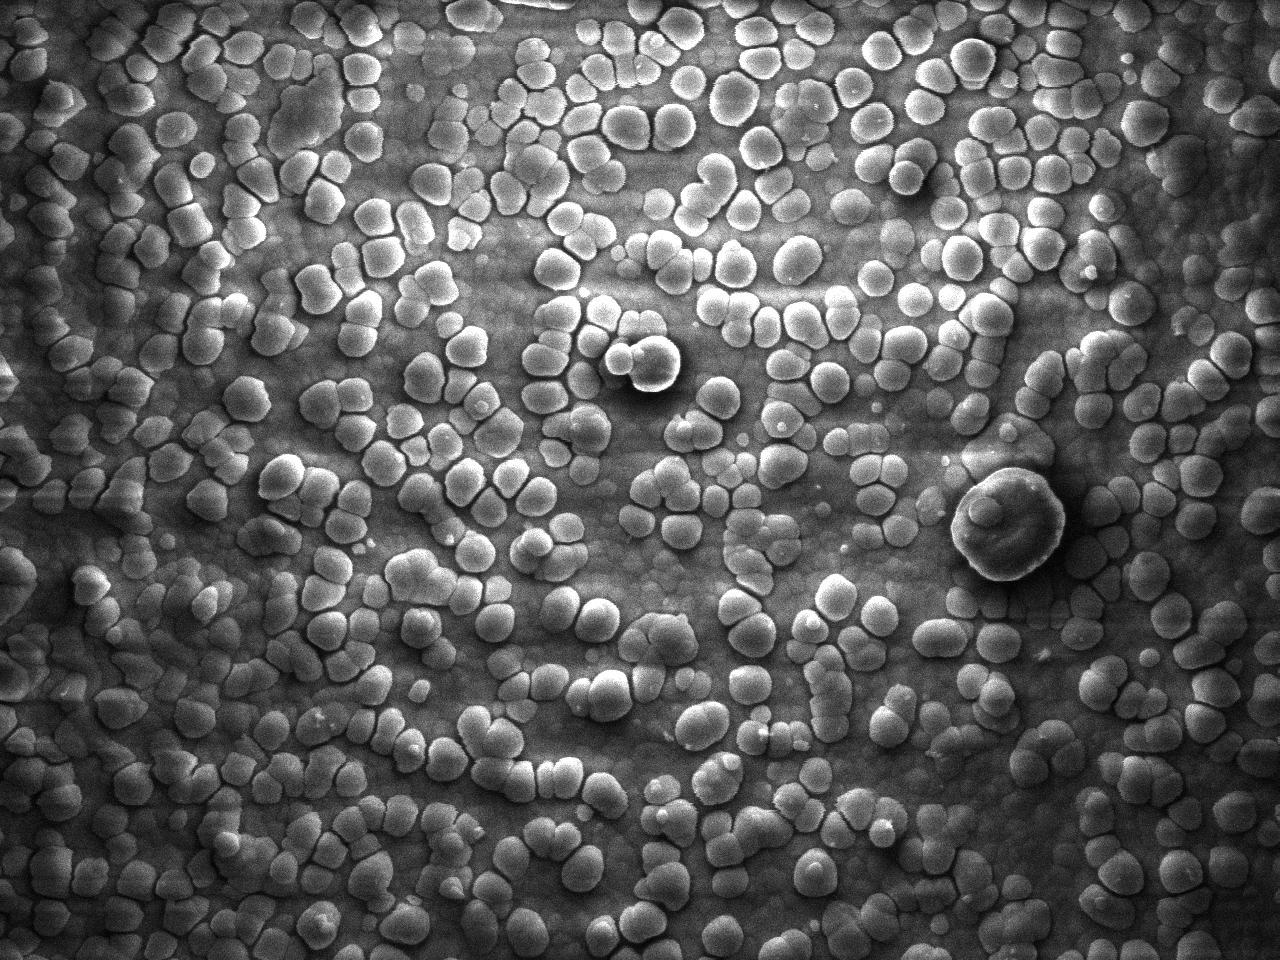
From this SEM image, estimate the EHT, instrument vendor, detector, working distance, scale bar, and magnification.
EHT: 3 kV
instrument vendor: JEOL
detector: SEI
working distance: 9.4 mm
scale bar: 1000 nm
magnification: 20 K X